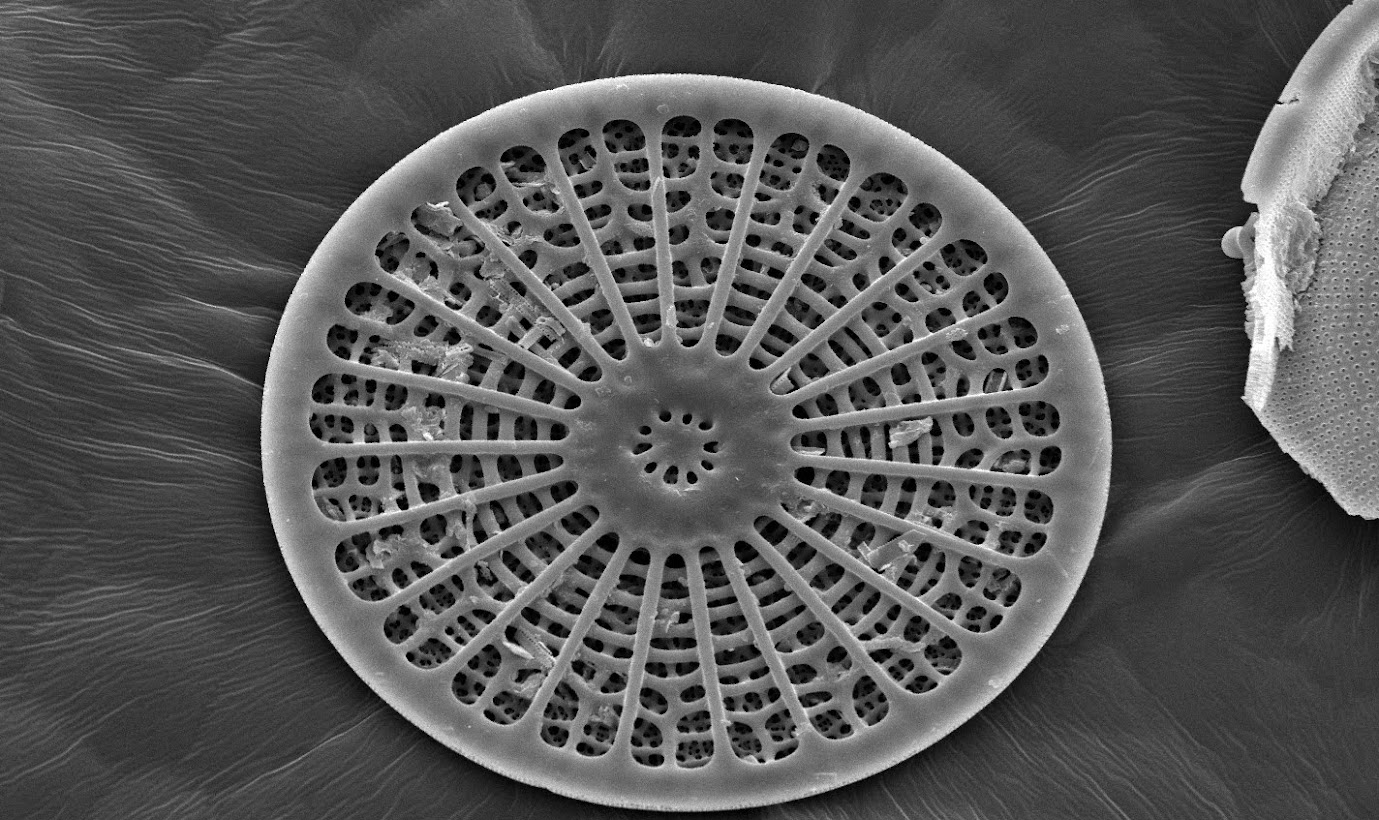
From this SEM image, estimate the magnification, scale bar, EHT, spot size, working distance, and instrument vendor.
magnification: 0.65 K X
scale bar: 100000 nm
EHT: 25 kV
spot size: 3.5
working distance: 15.2 mm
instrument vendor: FEI